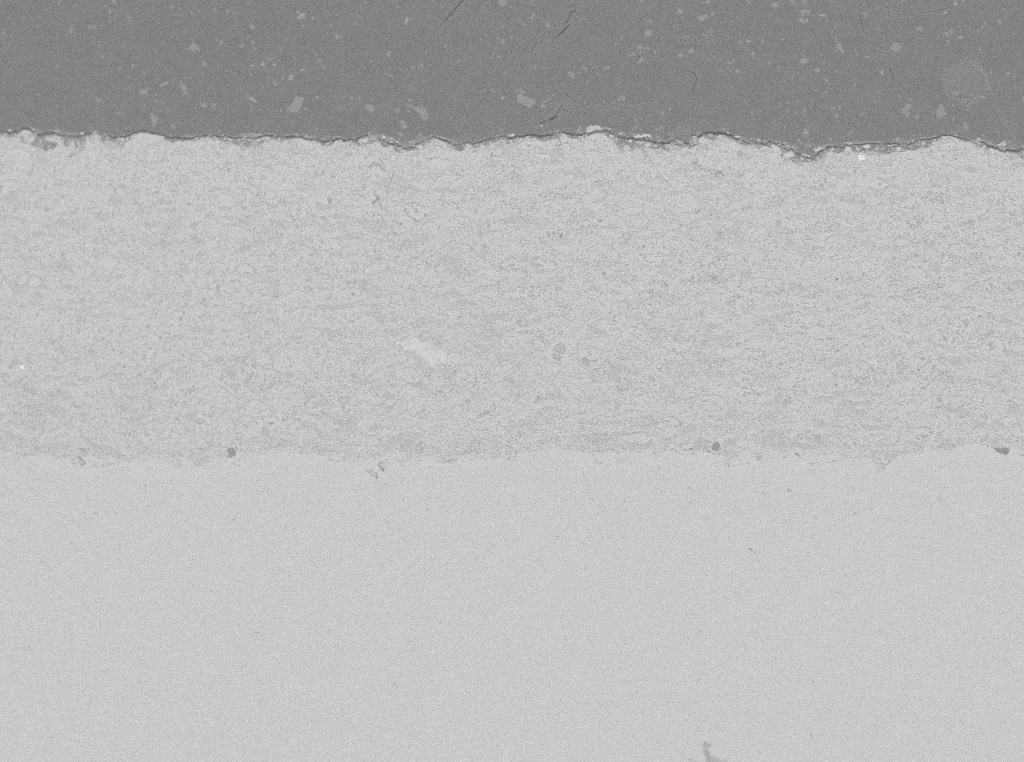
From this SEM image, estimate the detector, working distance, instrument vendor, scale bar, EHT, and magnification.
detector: vCD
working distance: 16.3 mm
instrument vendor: FEI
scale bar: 500000 nm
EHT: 15 kV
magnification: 0.25 K X